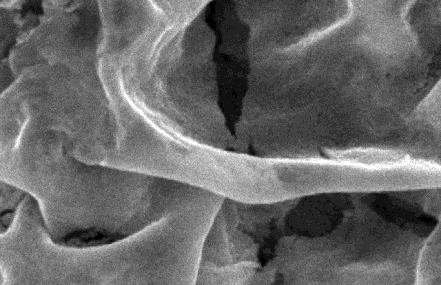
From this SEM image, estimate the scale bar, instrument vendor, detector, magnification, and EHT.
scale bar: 1000 nm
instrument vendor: JEOL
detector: SEI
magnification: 10 K X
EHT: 20 kV